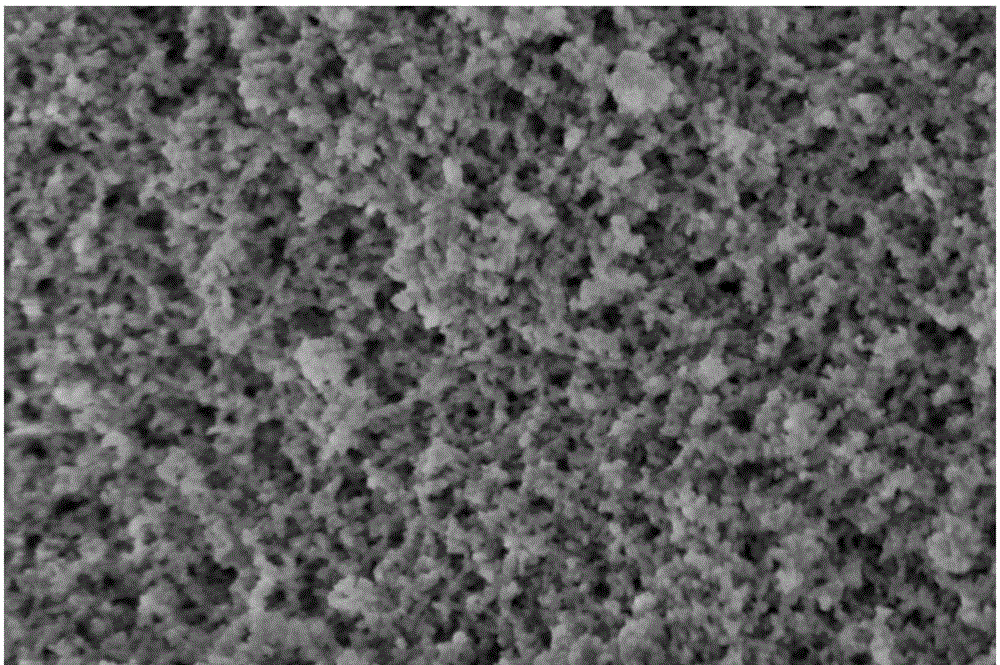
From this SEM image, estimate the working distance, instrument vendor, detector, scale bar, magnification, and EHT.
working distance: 2.5 mm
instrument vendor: FEI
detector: TLD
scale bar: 500 nm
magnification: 300 K X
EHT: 1 kV